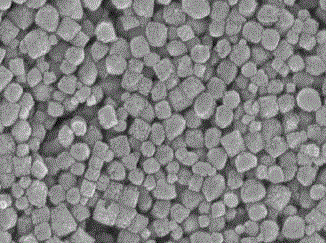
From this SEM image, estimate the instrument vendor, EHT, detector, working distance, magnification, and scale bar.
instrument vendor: JEOL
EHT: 10 kV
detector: SEI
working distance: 7 mm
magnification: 30 K X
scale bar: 100 nm